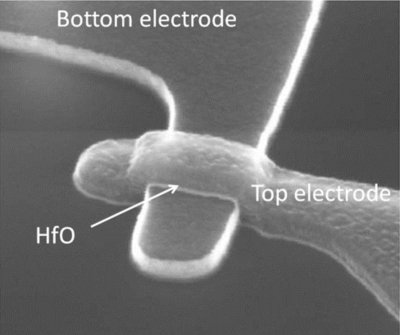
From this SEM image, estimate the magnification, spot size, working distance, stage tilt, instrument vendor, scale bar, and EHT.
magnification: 500 K X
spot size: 3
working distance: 5.005 mm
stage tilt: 50°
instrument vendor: FEI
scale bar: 100 nm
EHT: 15 kV